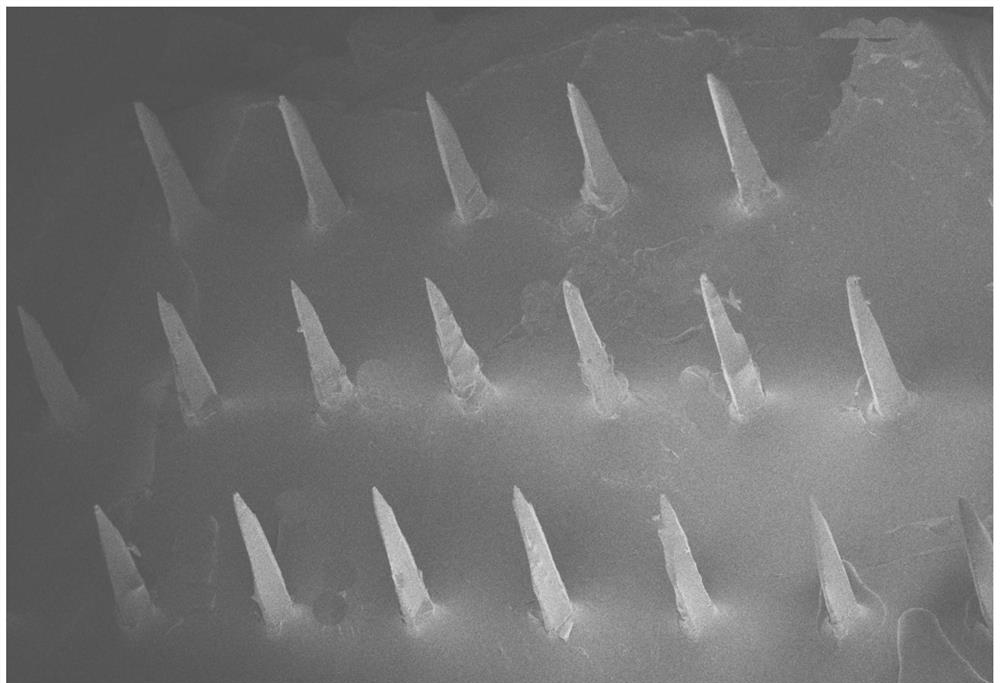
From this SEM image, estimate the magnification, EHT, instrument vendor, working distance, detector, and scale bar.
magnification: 0.03 K X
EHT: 5 kV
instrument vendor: Zeiss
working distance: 6.1 mm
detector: InLens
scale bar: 200000 nm